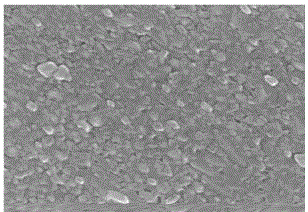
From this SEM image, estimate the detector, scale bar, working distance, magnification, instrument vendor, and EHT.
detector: SE(U)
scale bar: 1000 nm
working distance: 15 mm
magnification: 50 K X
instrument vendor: Hitachi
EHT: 15 kV